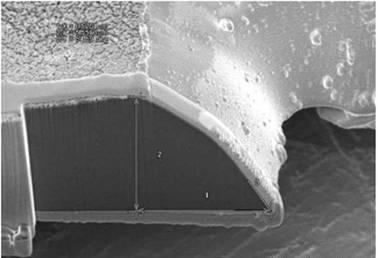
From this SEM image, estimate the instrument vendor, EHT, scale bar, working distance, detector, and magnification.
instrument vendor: Hitachi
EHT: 3 kV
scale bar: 5000 nm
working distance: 16.3 mm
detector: SE(M)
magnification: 7 K X